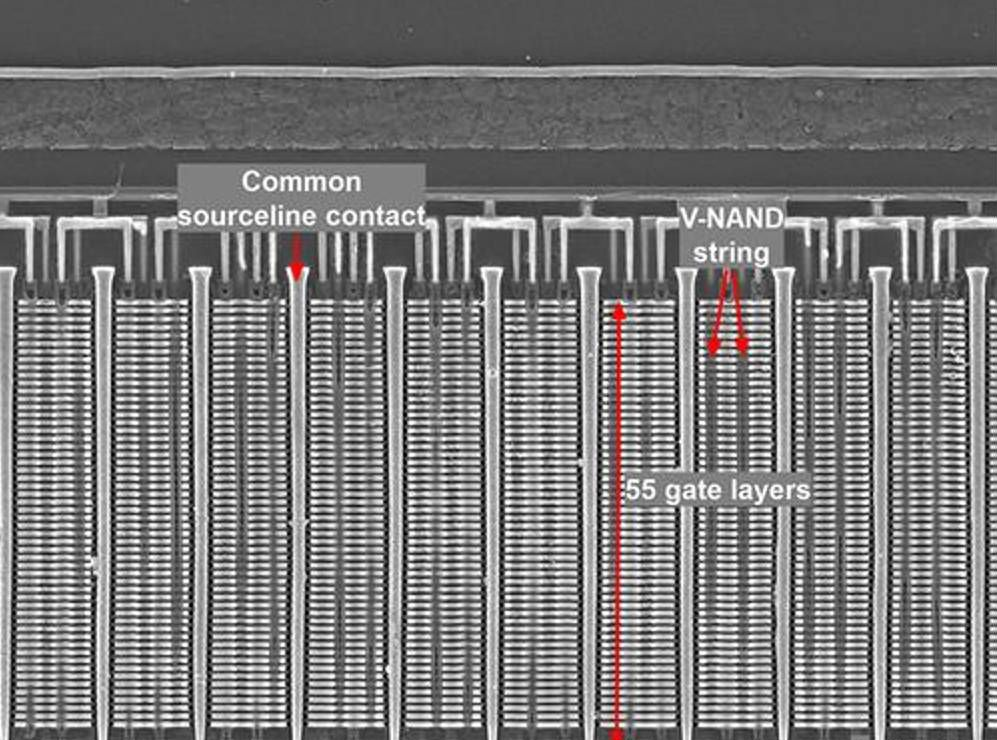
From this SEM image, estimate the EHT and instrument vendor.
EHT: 5 kV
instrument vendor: Zeiss